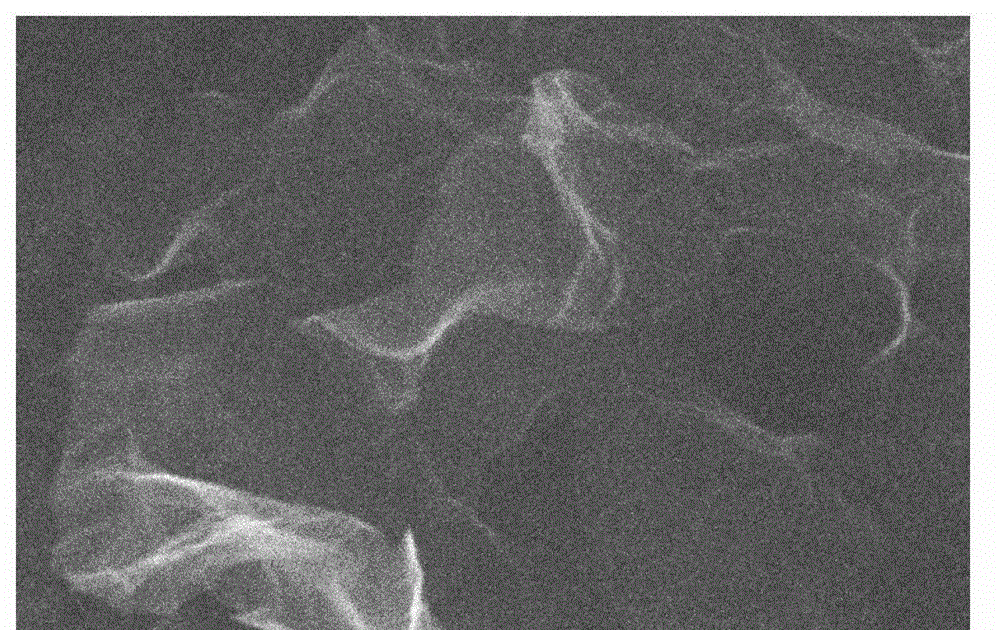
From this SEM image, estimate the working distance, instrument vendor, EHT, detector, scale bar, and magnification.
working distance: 5.2 mm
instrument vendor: Hitachi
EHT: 15 kV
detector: SE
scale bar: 2000 nm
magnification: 20 K X